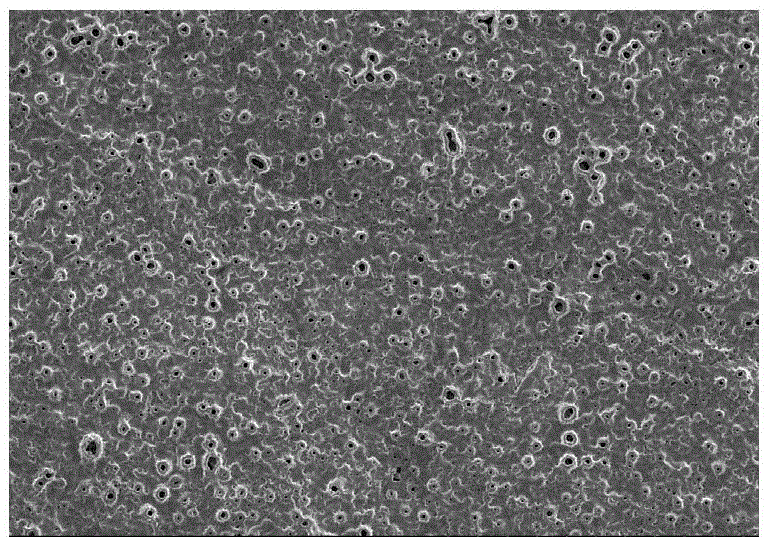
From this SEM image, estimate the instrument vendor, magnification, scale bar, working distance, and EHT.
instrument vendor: Hitachi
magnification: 0.5 K X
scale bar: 100000 nm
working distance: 10.7 mm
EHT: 5 kV